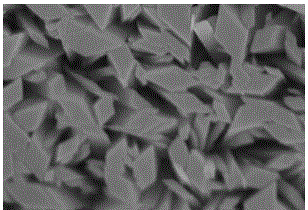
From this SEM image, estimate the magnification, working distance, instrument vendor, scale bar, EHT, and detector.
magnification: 50 K X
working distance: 15 mm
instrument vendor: Hitachi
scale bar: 1000 nm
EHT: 15 kV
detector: SE(U)